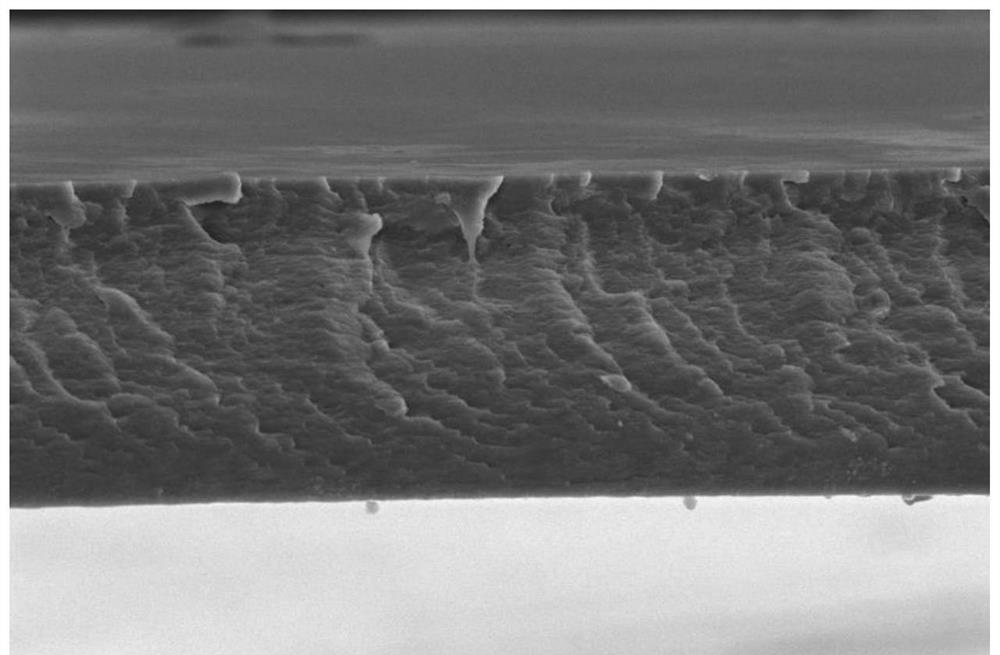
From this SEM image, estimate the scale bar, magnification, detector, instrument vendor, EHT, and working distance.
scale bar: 30000 nm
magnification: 4 K X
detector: ETD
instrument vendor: FEI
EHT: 10 kV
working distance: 5.2 mm